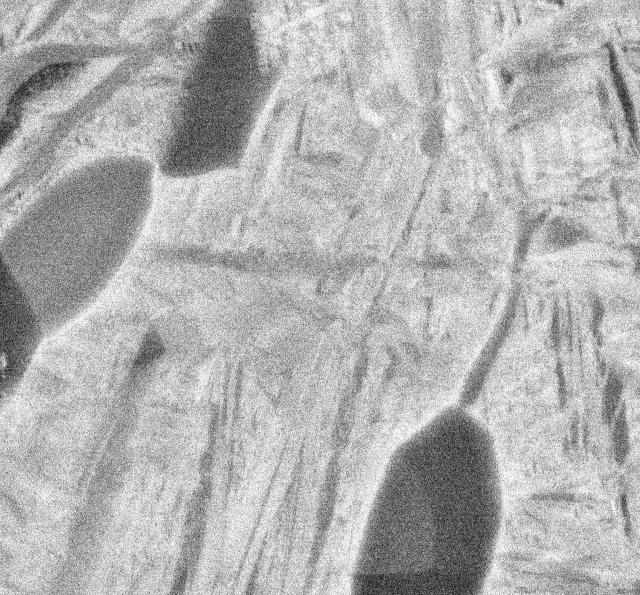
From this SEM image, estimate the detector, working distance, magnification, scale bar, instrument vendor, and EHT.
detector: COMPO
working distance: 10 mm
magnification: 10 K X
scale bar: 1000 nm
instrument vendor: JEOL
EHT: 15 kV